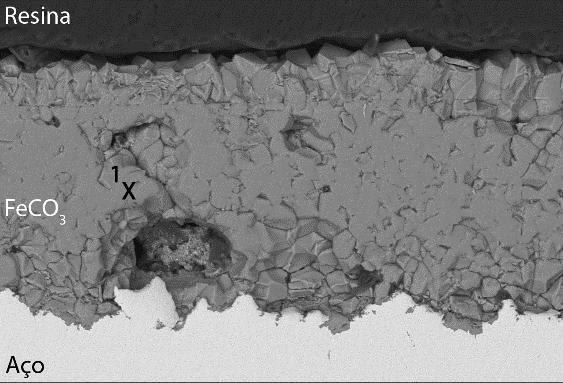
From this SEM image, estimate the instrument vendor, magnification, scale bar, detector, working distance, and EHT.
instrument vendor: JEOL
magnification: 0.5 K X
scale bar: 50000 nm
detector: BEC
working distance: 10 mm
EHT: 15 kV